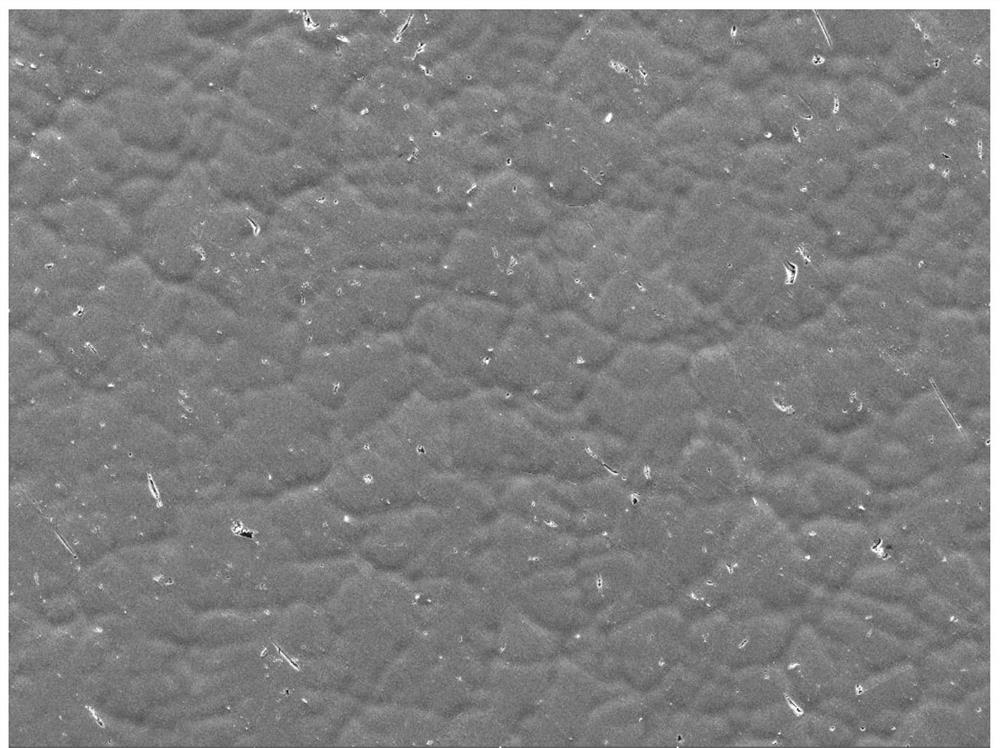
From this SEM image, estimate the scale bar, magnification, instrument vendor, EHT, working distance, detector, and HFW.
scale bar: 100000 nm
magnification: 0.5 K X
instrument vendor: Tescan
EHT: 20 kV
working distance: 14.75 mm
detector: SE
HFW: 554 µm